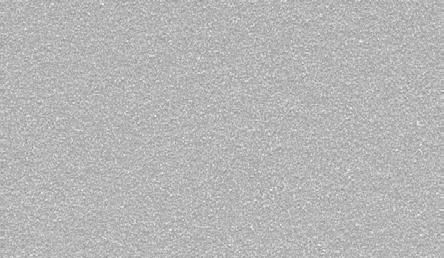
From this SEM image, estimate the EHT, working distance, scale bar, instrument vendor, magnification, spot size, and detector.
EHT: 25 kV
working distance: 9.7 mm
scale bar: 50000 nm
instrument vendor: FEI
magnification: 1 K X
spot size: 4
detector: SE